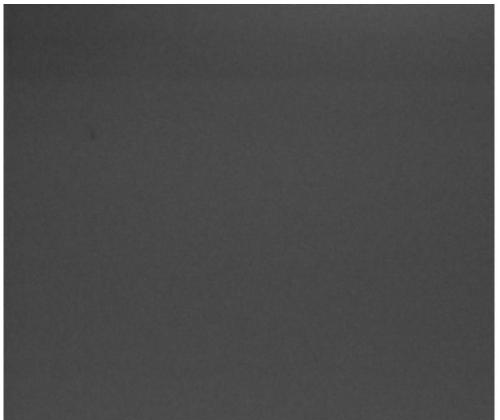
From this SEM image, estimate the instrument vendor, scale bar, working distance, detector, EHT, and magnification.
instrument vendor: FEI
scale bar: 500 nm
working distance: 4.8 mm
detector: TLD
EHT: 10 kV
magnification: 120 K X